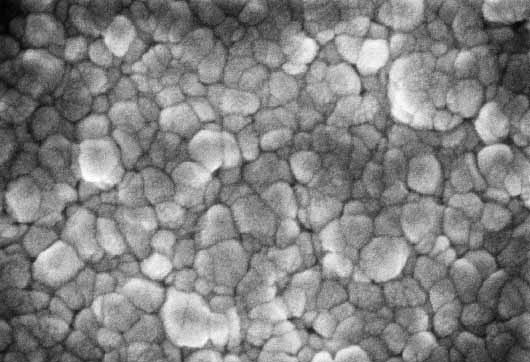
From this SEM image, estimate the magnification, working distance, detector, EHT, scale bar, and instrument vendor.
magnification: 50 K X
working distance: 3.2 mm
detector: InLens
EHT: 3 kV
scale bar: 100 nm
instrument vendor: Zeiss